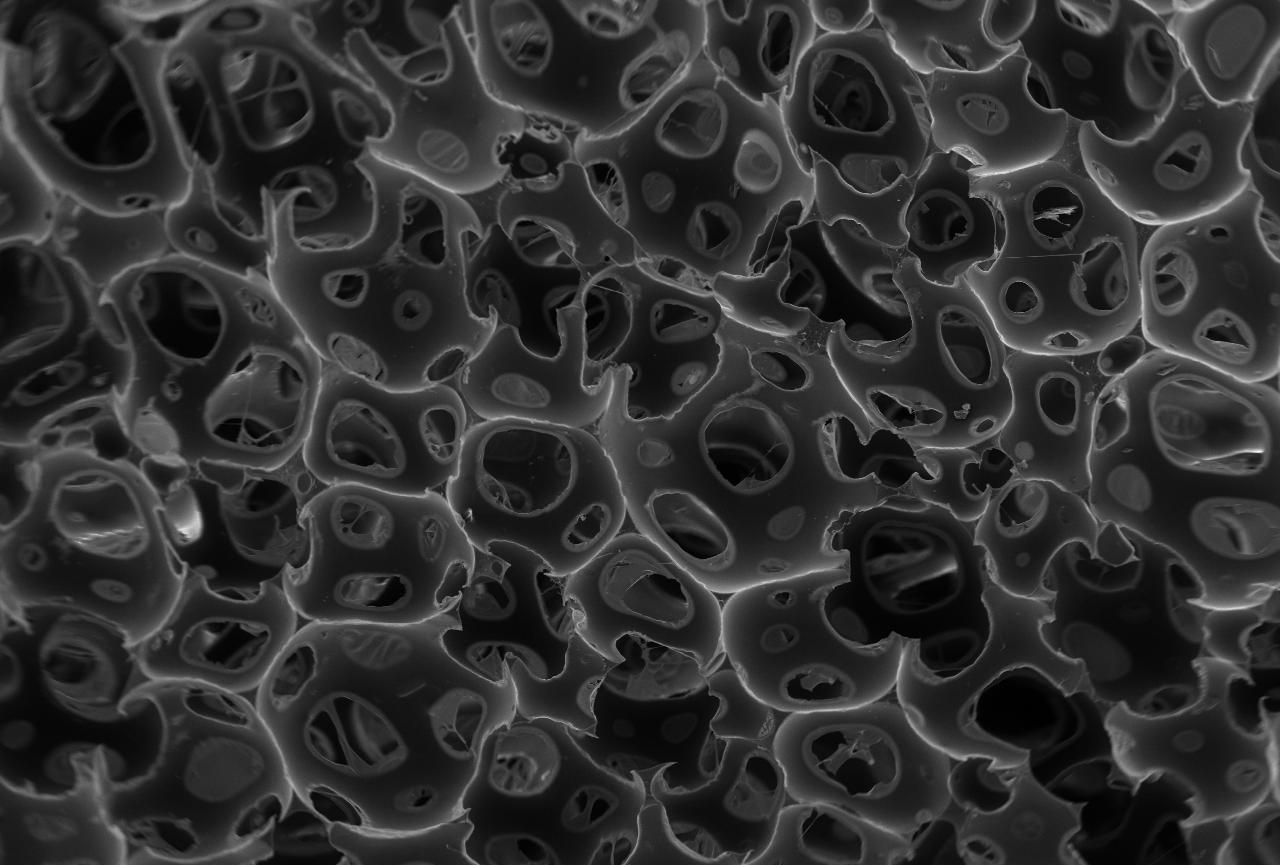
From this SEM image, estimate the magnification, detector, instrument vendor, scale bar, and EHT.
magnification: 0.05 K X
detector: SEI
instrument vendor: JEOL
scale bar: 500000 nm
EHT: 15 kV